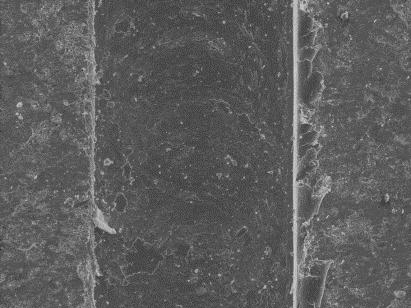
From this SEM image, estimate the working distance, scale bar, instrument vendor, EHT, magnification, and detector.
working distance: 29 mm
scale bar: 100000 nm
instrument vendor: Zeiss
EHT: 20 kV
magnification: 0.25 K X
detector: SE1